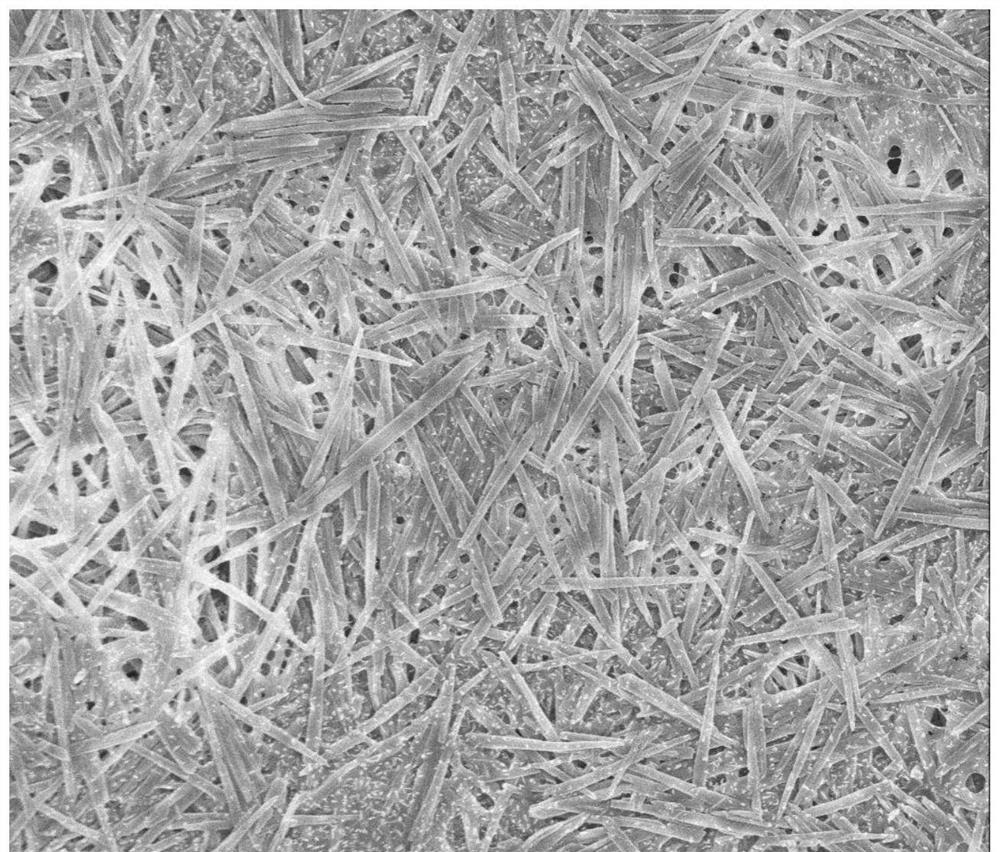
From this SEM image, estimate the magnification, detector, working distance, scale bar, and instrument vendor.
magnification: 8 K X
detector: TLD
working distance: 4.6 mm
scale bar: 5000 nm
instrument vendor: FEI